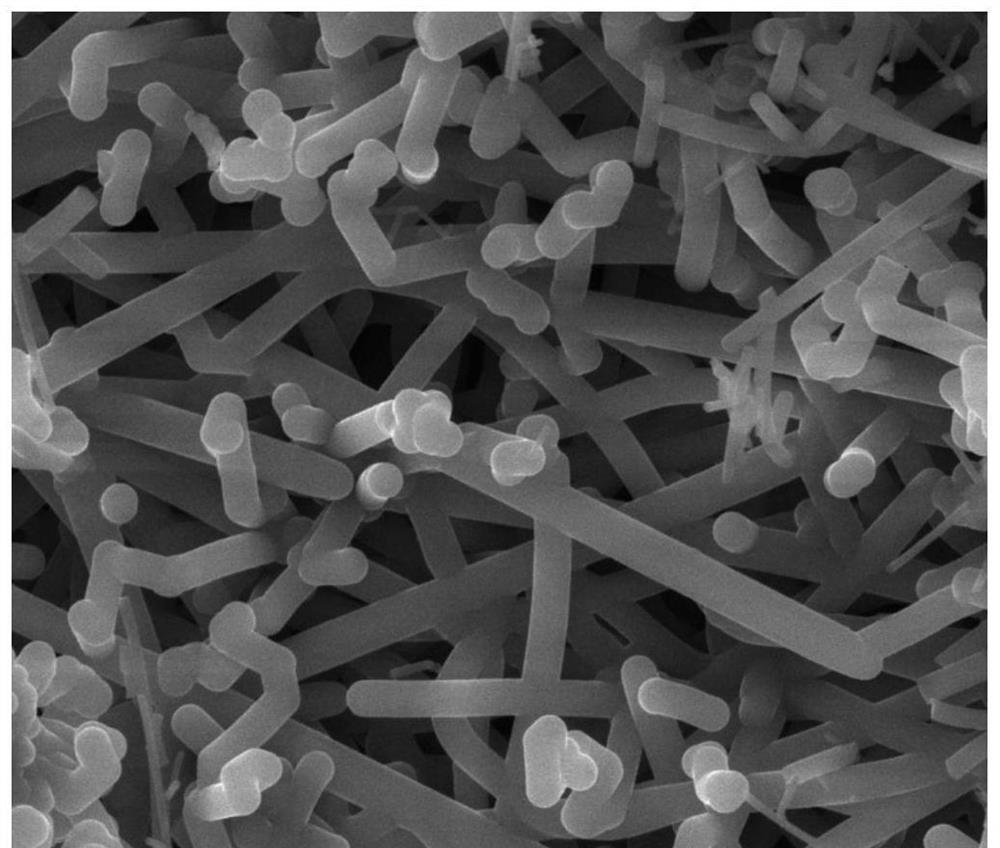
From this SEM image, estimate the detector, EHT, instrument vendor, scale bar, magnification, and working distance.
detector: ETD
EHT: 25 kV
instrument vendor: FEI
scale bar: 10000 nm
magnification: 5 K X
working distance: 17.9 mm